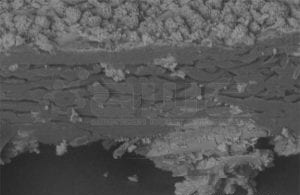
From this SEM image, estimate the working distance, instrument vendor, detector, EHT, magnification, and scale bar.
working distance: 40 mm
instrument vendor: Hitachi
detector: BSE3D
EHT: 15 kV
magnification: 0.42 K X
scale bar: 100000 nm